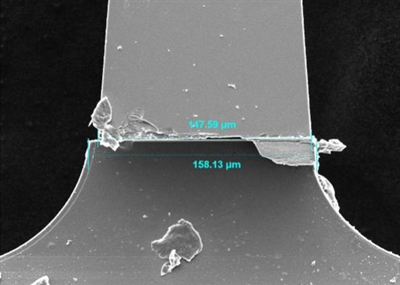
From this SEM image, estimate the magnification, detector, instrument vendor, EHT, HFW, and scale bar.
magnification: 1 K X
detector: SE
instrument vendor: Tescan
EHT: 10 kV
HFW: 277 µm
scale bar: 50000 nm